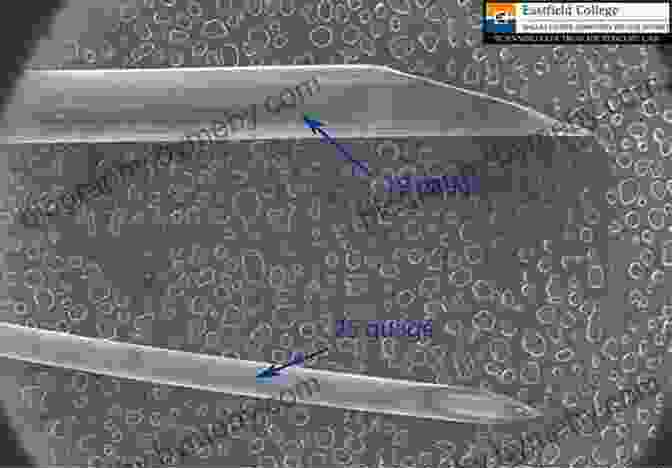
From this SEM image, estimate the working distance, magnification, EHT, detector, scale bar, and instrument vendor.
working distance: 25.6 mm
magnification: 0.011 K X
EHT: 14 kV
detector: SE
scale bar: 5000000 nm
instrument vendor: Hitachi